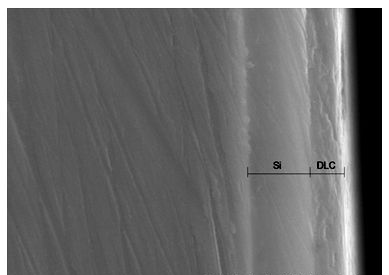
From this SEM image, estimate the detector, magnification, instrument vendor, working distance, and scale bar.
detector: SE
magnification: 10 K X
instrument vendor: JEOL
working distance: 6.3 mm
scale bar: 5000 nm